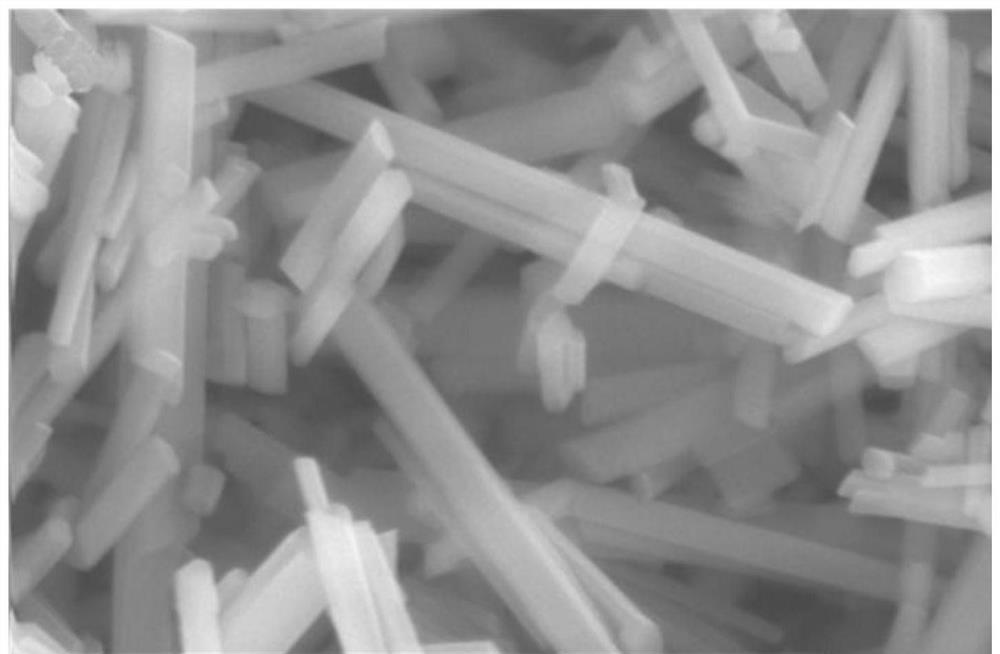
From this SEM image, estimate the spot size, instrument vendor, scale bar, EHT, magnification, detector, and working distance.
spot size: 4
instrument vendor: FEI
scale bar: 2000 nm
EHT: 20 kV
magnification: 20 K X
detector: SE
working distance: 5.5 mm